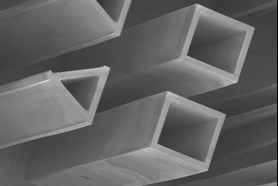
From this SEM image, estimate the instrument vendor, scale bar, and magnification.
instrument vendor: Hitachi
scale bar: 600000 nm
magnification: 0.05 K X